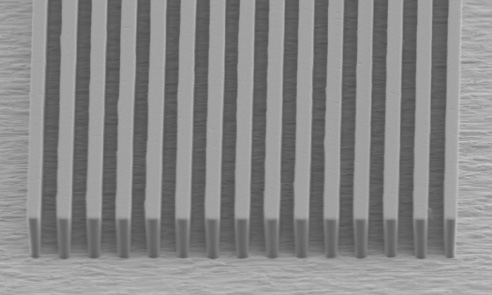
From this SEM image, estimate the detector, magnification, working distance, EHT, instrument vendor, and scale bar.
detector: SEI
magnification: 0.8 K X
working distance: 21 mm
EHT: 5 kV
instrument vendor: JEOL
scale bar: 20000 nm